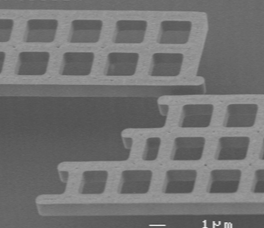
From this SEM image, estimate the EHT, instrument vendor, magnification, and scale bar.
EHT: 2 kV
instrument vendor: JEOL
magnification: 5 K X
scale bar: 1000 nm